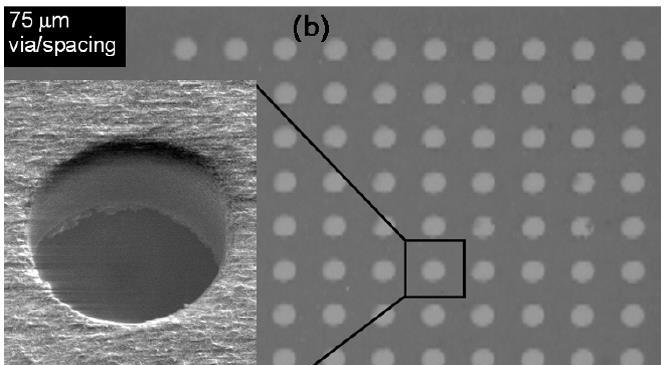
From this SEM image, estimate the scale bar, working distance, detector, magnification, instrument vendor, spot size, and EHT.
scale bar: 50000 nm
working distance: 9.1 mm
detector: GSE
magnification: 0.5 K X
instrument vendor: FEI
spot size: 3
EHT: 10 kV